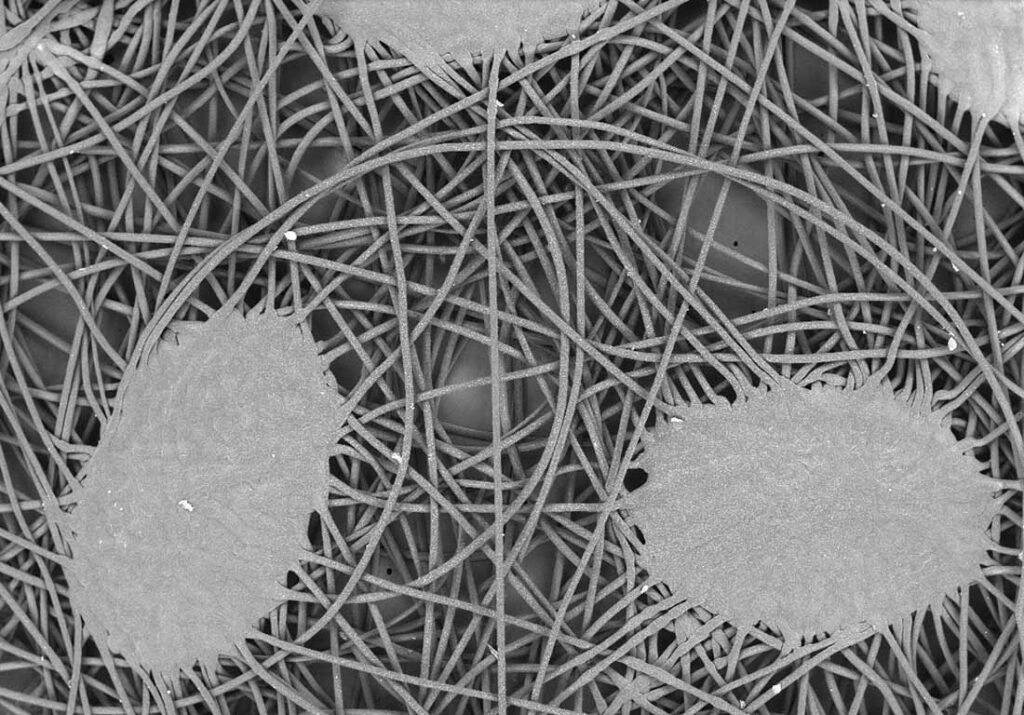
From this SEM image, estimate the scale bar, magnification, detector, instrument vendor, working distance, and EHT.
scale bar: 1000000 nm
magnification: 0.05 K X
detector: BSE-COMP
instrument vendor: Hitachi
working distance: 7.3 mm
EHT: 10 kV